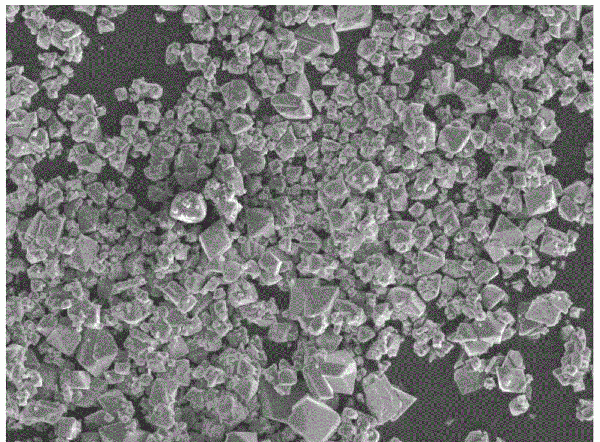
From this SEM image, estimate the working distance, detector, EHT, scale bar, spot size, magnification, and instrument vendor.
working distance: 11 mm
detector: SED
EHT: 15 kV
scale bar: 50000 nm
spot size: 30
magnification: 0.5 K X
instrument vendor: JEOL